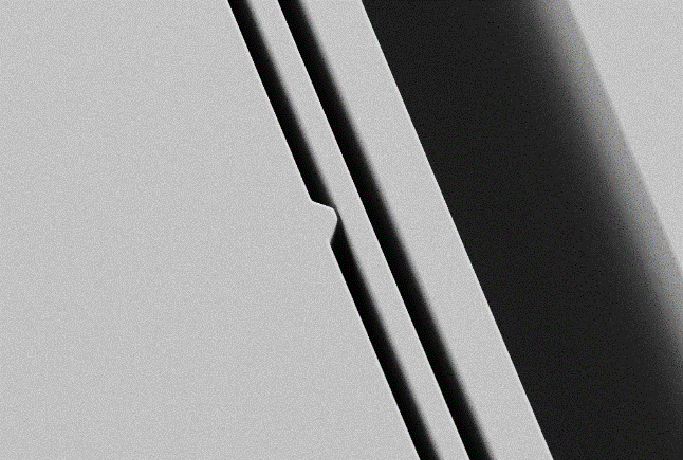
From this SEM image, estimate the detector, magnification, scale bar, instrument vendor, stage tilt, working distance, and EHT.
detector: SE2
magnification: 1 K X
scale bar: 20000 nm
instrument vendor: Zeiss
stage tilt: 25°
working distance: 6.1 mm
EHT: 1 kV